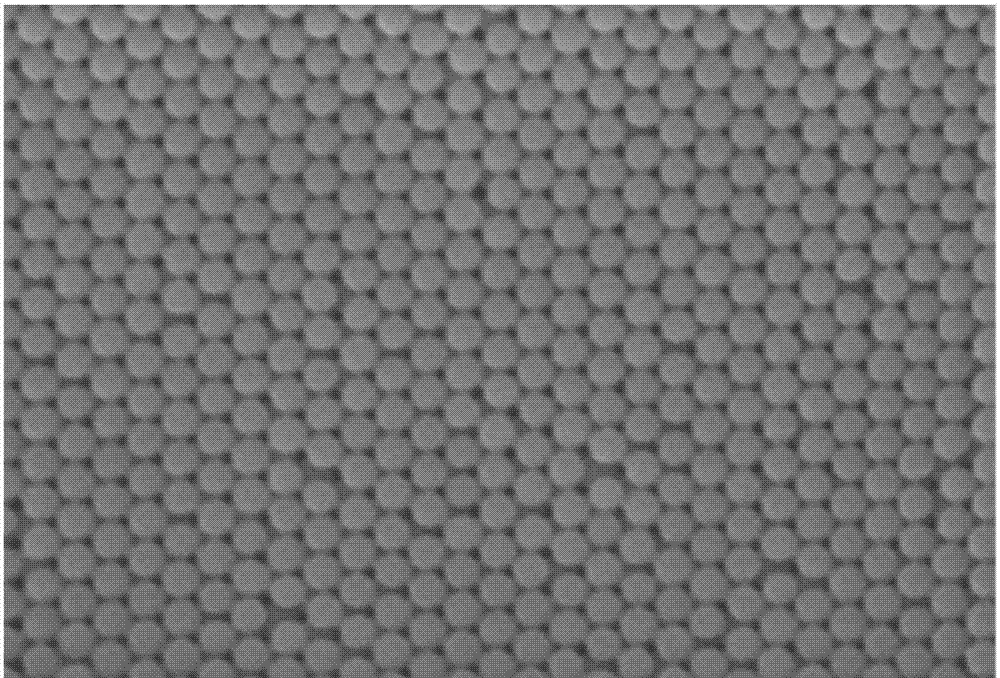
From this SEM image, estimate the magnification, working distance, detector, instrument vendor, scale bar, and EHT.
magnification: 22 K X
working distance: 10.4 mm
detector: SE(M)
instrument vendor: Hitachi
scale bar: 2000 nm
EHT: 5 kV